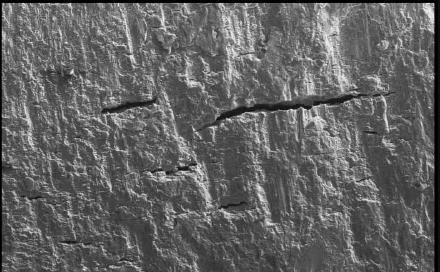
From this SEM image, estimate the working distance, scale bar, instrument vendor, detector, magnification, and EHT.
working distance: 25 mm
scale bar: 200000 nm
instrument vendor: Zeiss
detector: SE1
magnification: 0.225 K X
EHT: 20 kV